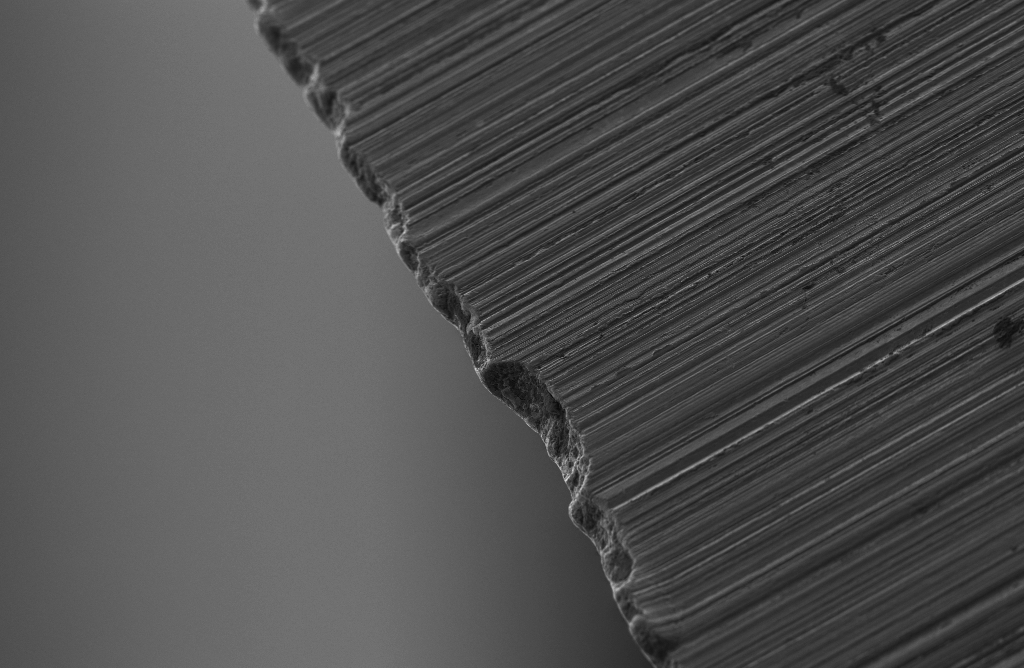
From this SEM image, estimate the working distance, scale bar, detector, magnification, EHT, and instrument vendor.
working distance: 7.3 mm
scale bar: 20000 nm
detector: SE2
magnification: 0.2 K X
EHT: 3 kV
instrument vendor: Zeiss